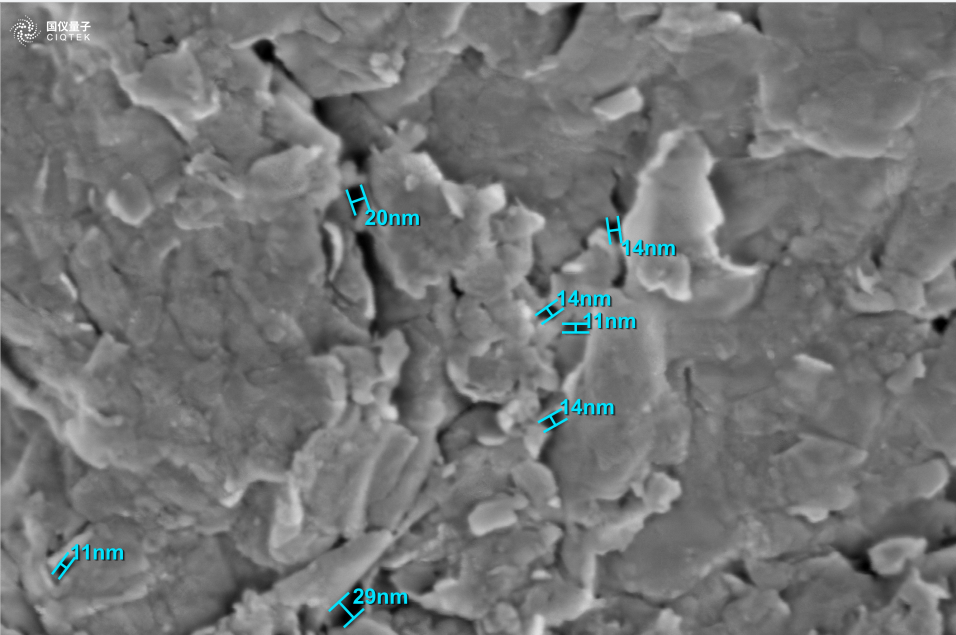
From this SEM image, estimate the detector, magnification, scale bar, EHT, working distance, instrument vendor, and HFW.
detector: INLENS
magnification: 100 K X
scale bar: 100 nm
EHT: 1 kV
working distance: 3.38 mm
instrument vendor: other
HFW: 1.28 µm